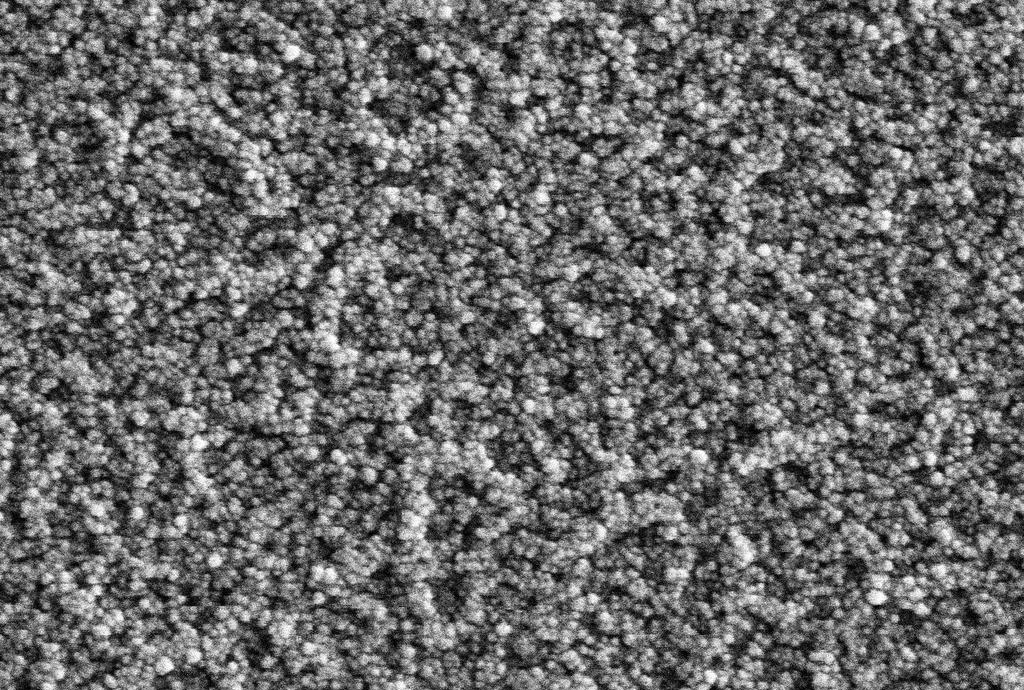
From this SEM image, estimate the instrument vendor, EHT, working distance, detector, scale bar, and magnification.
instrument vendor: Hitachi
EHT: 7 kV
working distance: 7.5 mm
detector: SE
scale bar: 500 nm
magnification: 60 K X